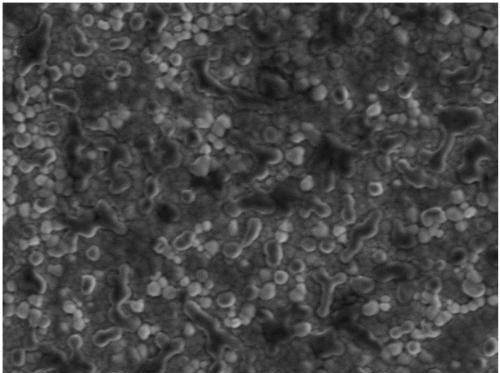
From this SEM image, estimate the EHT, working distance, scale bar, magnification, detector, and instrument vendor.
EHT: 2 kV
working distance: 4.3 mm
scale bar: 100 nm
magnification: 30 K X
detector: UED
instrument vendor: JEOL